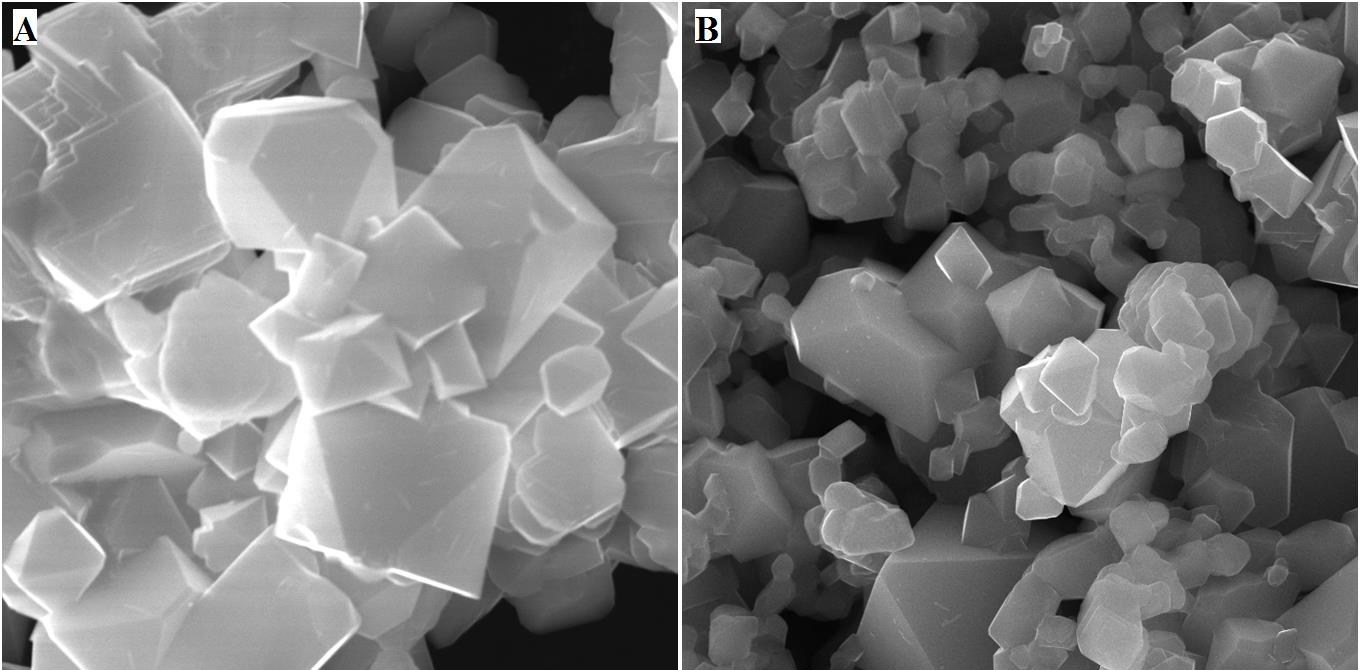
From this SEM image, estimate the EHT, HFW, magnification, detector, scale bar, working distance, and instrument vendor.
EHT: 30 kV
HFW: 13.8 µm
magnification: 15 K X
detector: SE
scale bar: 2000 nm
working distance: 15 mm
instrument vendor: Tescan